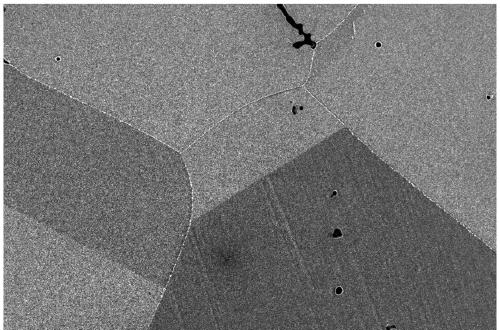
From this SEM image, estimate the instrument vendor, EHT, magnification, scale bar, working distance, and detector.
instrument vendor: Zeiss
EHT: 5 kV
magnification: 1 K X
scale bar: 10000 nm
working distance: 6.7 mm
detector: InLens